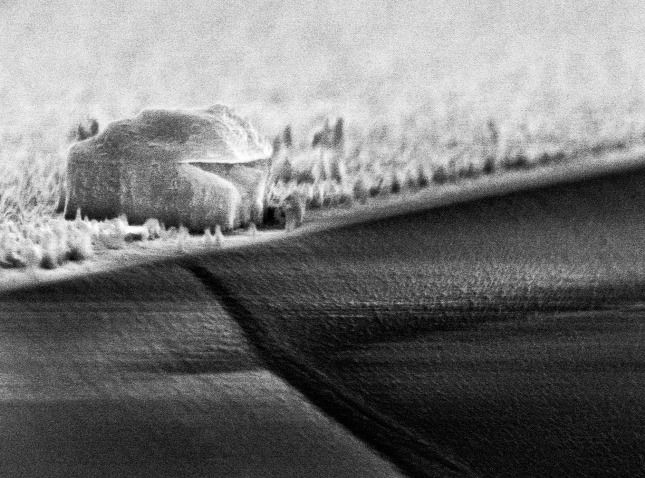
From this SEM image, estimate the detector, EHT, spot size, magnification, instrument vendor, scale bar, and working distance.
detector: TLD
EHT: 10 kV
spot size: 2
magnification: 24.008 K X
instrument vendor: FEI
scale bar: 1000 nm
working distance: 5.8 mm